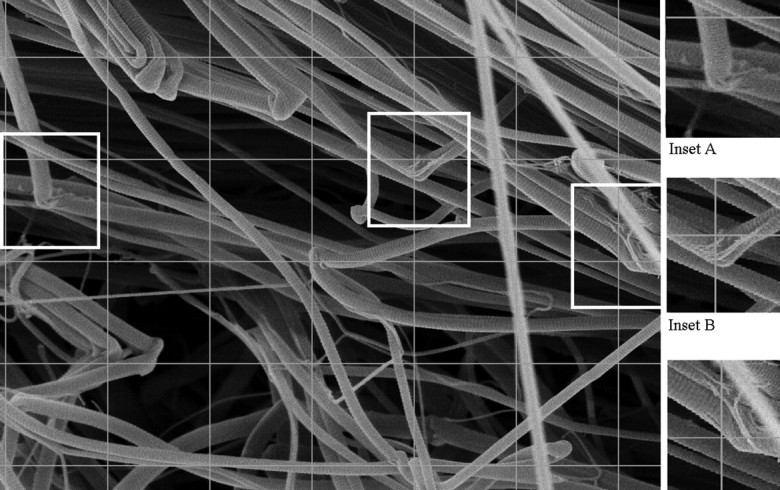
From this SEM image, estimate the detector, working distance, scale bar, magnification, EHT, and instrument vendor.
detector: InLens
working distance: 5 mm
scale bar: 300 nm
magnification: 30.35 K X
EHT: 5 kV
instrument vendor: Zeiss